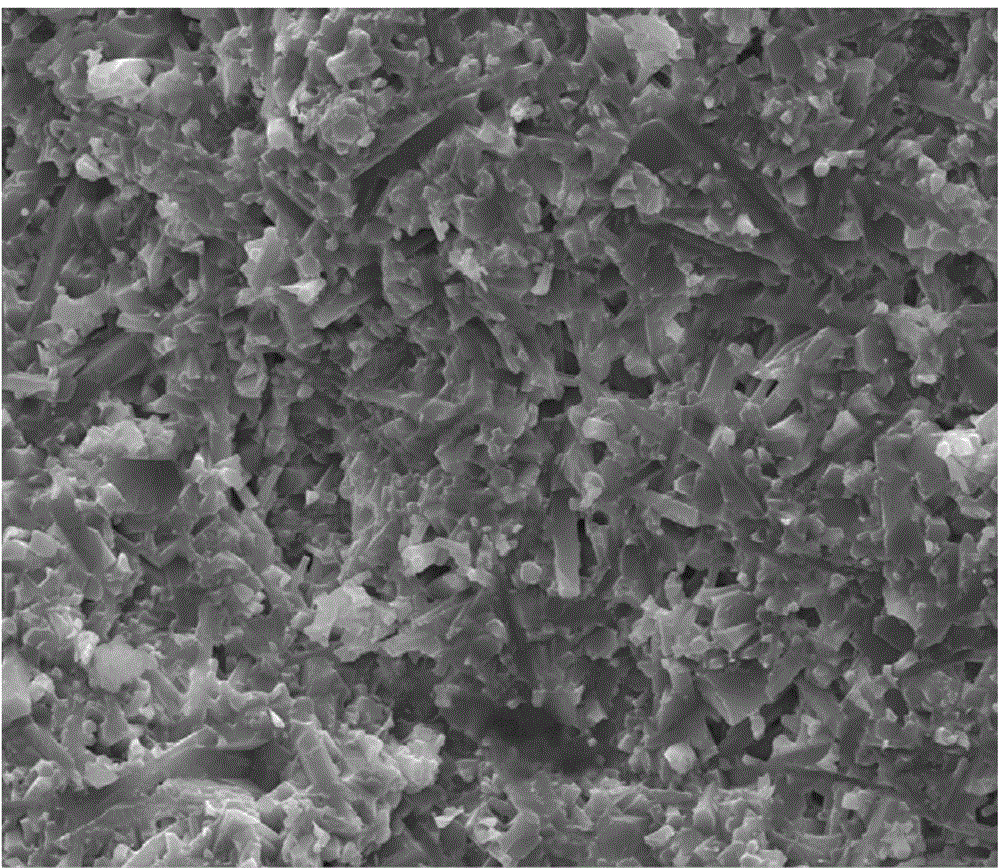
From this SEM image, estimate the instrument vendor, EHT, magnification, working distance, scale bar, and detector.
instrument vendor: FEI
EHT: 20 kV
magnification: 5 K X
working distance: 9.1 mm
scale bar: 20000 nm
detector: SE